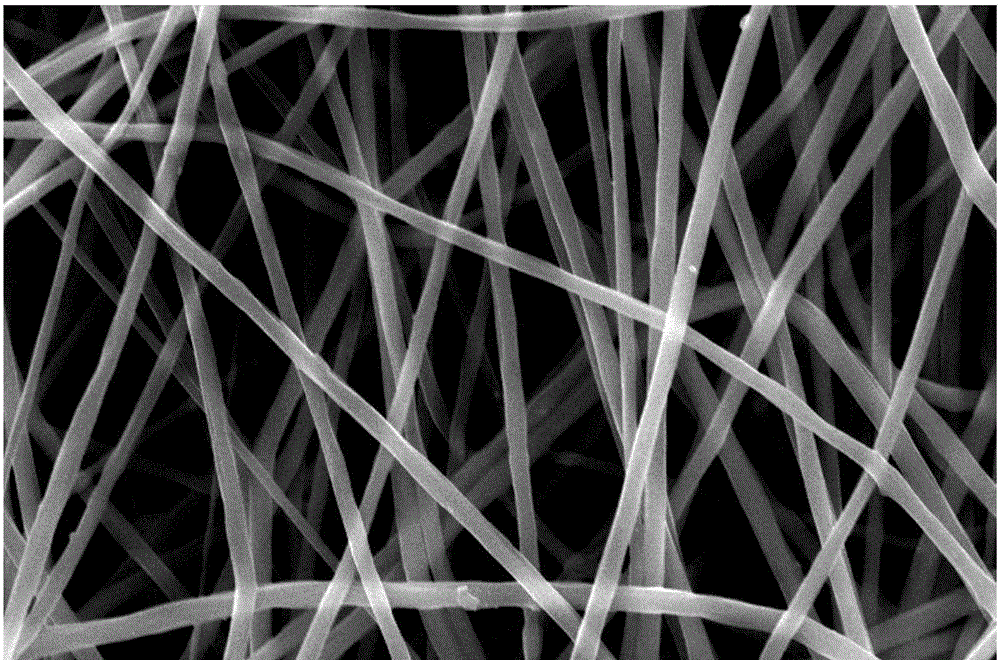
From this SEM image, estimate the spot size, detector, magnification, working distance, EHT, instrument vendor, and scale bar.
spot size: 3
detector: ETD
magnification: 6 K X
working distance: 13.8 mm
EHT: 20 kV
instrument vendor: FEI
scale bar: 20000 nm